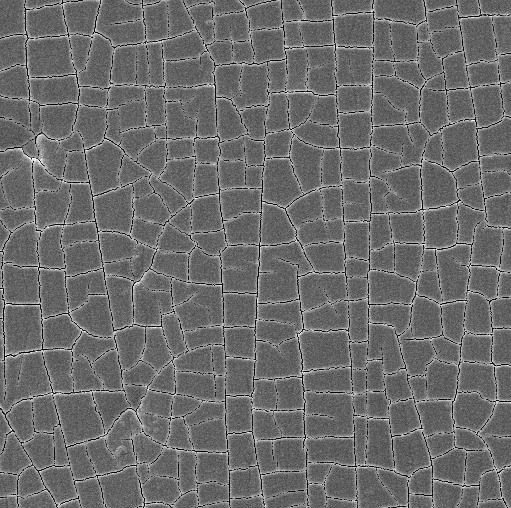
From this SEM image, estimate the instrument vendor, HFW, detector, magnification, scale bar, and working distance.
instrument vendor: Tescan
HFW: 138 µm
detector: SE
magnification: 1 K X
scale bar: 20000 nm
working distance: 15.19 mm